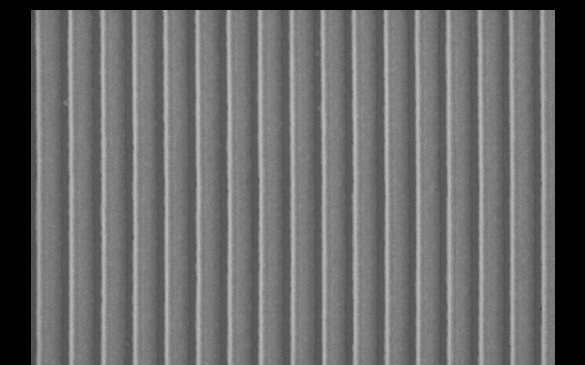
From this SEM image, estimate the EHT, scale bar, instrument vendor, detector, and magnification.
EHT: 0.5 kV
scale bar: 1000 nm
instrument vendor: Zeiss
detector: InLens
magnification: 16.94 K X